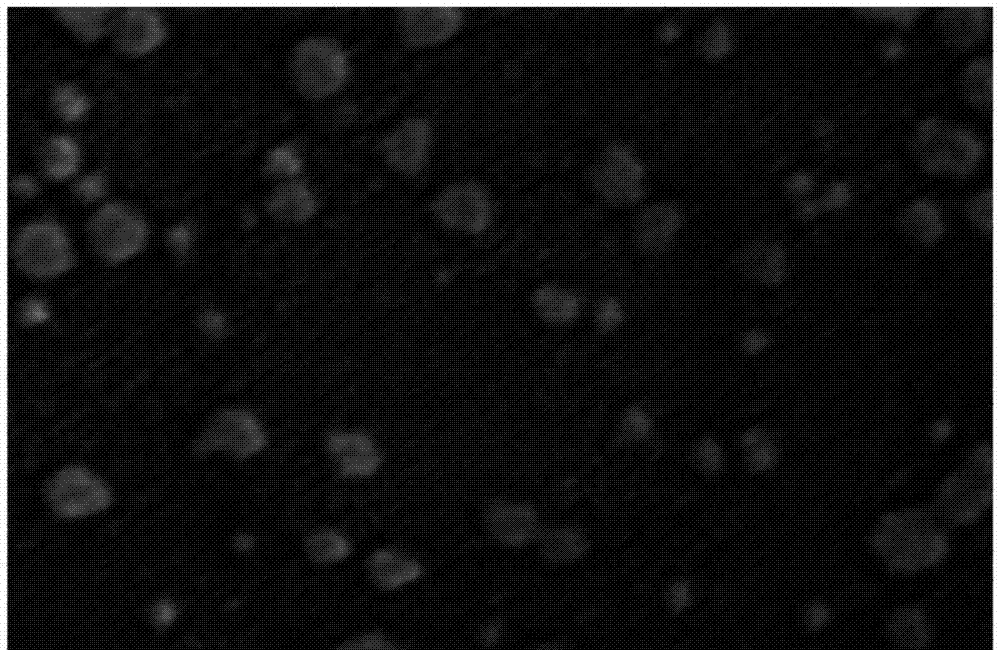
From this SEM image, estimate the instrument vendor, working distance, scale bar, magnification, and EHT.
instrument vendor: Hitachi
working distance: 9 mm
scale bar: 300 nm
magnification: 180 K X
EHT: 3 kV